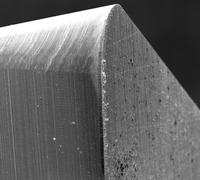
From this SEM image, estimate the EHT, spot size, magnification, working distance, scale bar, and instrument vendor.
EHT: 25 kV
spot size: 4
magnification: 0.1 K X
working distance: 21.4 mm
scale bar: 500000 nm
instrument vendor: FEI